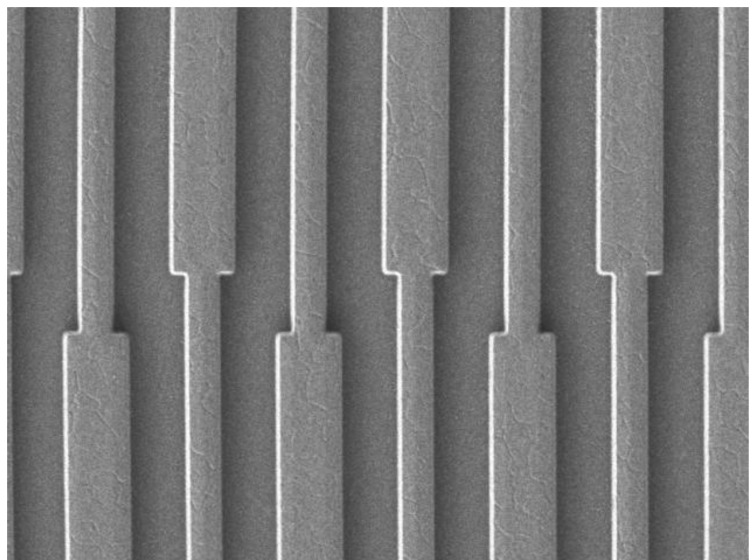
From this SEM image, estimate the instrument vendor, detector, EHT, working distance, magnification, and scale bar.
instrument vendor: JEOL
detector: LEI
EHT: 3 kV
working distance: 6.8 mm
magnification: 1 K X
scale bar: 10000 nm